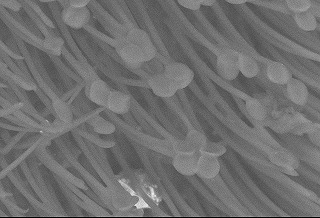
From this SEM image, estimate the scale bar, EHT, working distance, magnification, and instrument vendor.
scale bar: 30000 nm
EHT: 15 kV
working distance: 13.9 mm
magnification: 1.2 K X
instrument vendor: Hitachi